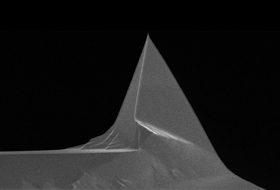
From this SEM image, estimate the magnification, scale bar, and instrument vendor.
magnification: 4.5 K X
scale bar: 5000 nm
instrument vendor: FEI